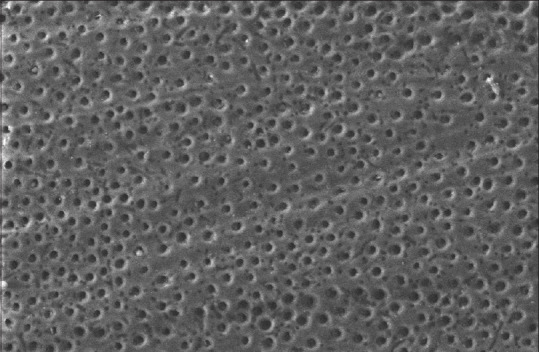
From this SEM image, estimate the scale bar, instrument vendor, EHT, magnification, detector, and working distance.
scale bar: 10000 nm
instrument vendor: Zeiss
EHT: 15 kV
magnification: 1.5 K X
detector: VPSE G3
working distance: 6 mm